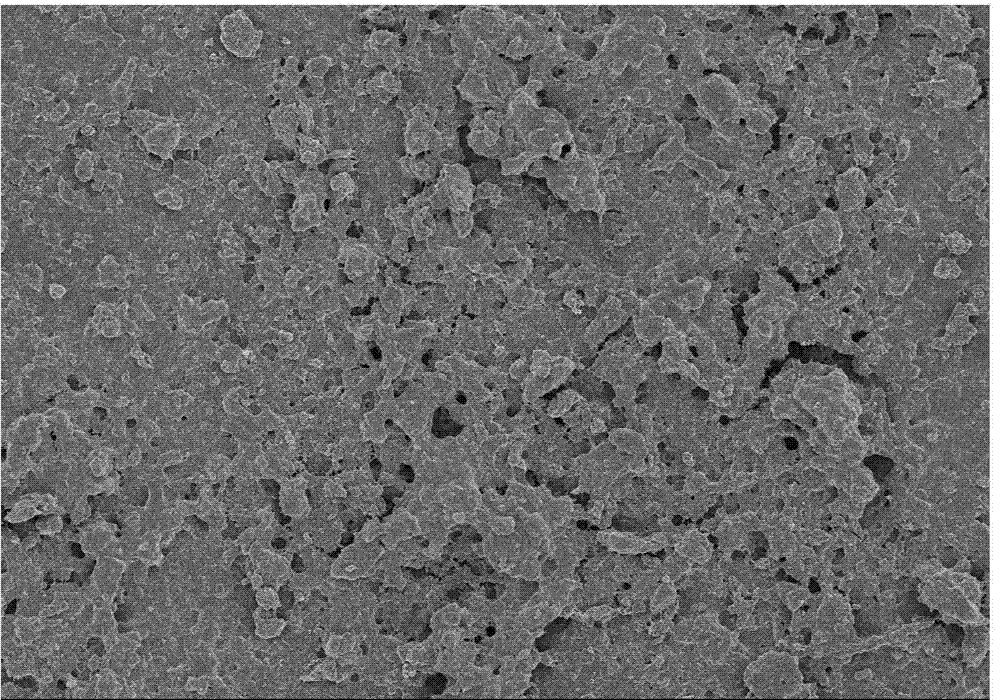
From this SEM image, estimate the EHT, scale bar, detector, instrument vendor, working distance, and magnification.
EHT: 3 kV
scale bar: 50000 nm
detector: SE(M)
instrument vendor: Hitachi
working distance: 8.5 mm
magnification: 1 K X